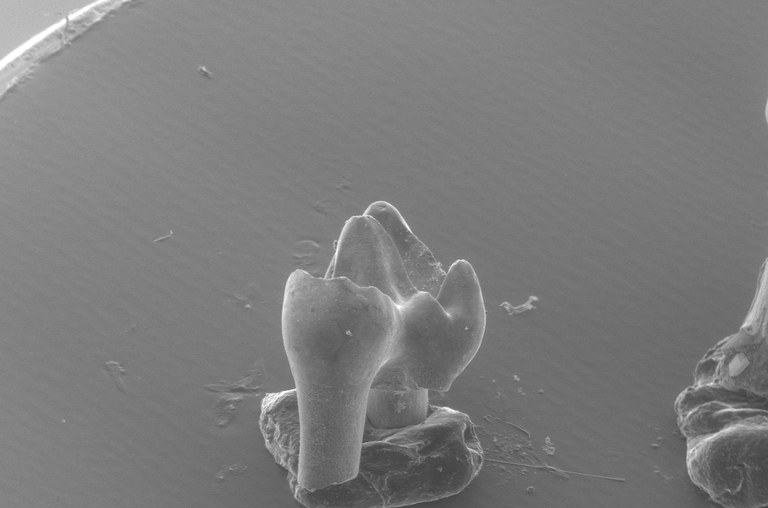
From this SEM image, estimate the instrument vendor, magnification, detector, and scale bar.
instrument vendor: FEI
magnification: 0.045 K X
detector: AUX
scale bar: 1000000 nm